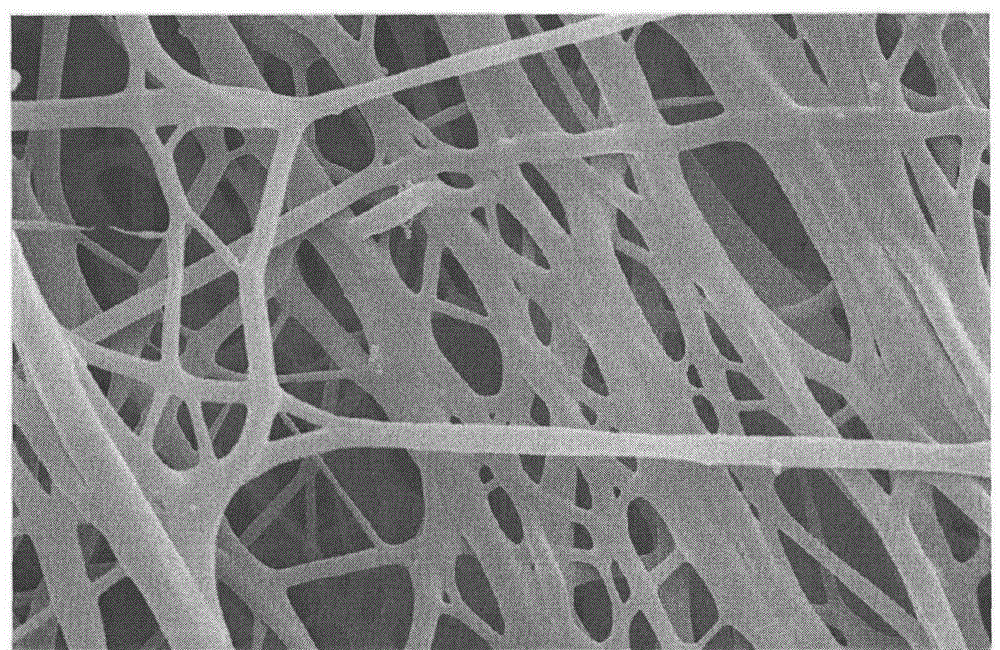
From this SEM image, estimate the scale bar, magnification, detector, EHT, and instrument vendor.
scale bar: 5000 nm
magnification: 8 K X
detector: SE(M)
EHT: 10 kV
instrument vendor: Hitachi